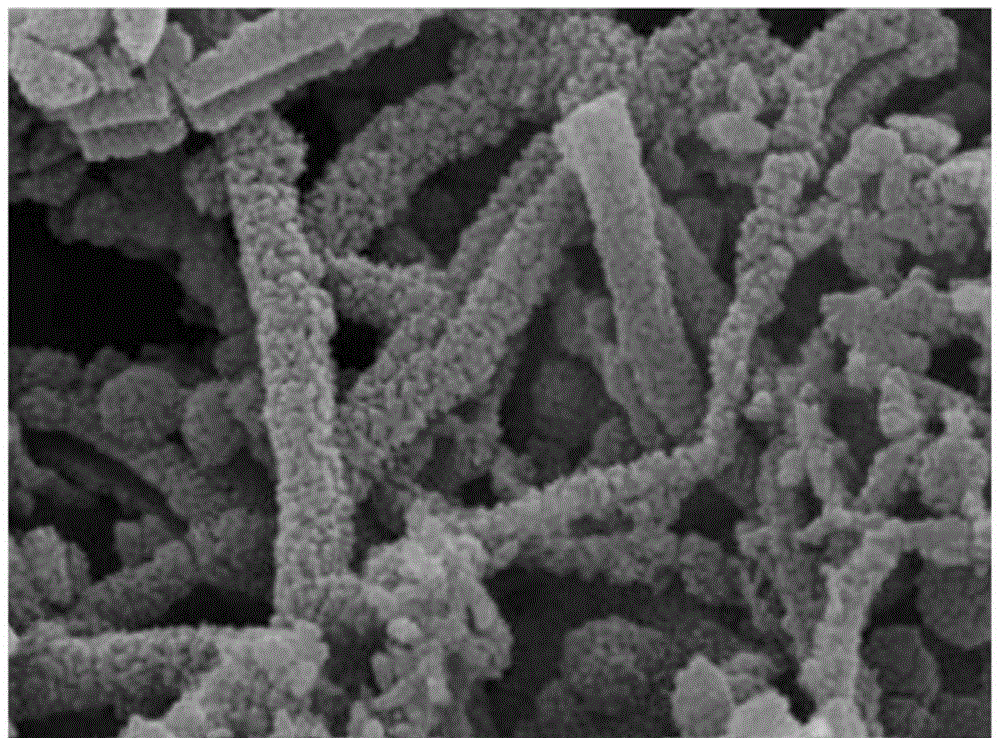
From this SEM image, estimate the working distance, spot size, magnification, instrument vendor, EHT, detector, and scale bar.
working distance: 4.9 mm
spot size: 3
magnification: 100 K X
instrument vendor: FEI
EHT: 15 kV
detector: TLD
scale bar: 500 nm